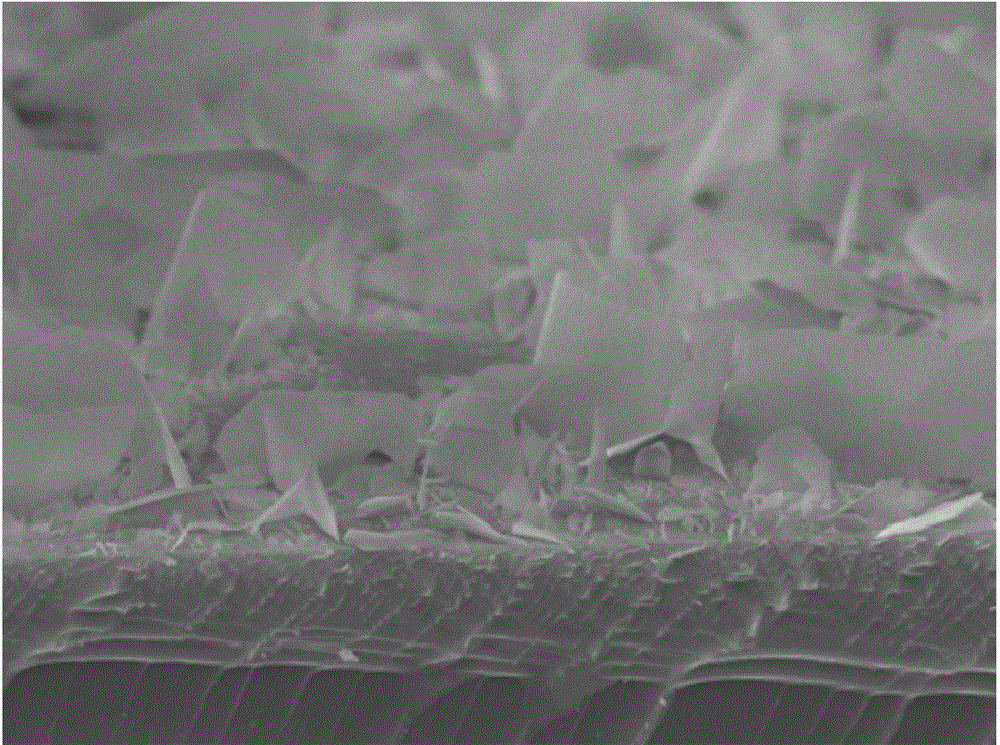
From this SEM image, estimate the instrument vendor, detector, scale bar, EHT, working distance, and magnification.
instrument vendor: JEOL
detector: SEI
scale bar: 1000 nm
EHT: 8 kV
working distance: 5.5 mm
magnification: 11 K X